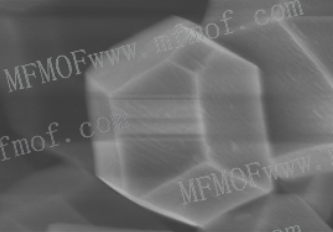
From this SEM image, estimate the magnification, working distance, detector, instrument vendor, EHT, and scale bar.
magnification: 60 K X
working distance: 8.4 mm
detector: SE(U)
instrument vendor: Hitachi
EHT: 3 kV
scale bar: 500 nm